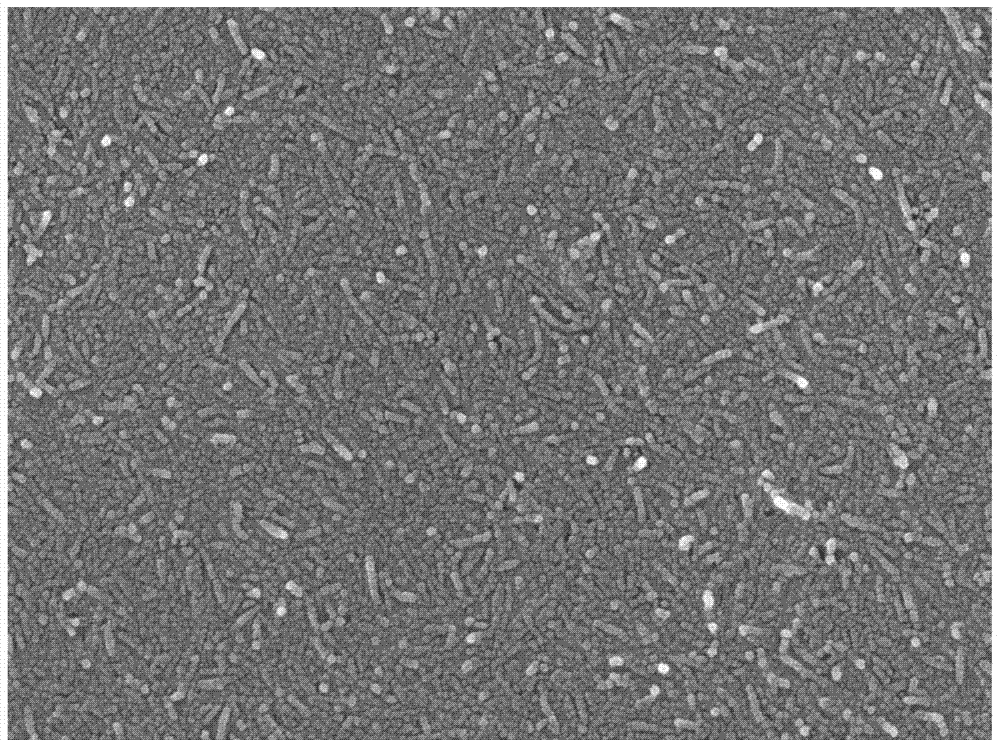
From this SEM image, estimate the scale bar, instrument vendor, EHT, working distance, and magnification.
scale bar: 100 nm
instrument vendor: JEOL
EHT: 10 kV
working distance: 6.8 mm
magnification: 50 K X